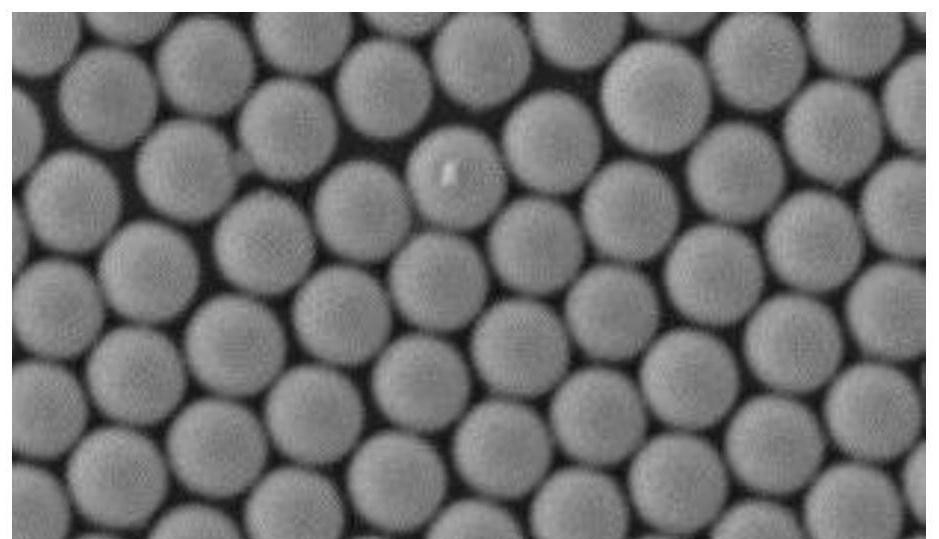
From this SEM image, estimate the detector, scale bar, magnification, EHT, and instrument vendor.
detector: SEI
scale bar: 1000 nm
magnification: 20 K X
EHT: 15 kV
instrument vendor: JEOL